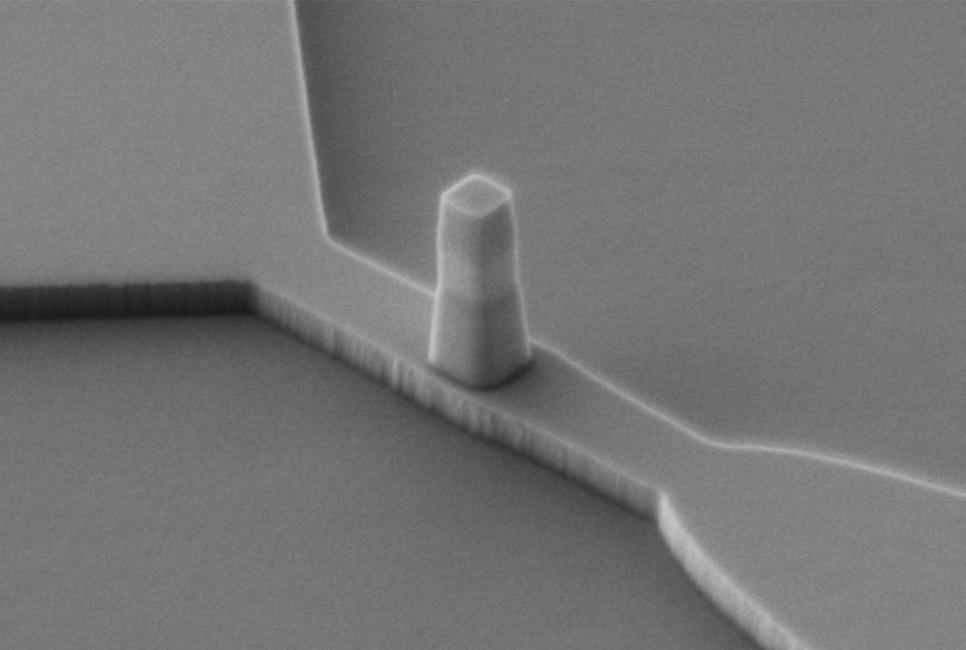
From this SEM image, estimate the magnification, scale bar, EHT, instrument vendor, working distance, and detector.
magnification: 23 K X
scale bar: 1000 nm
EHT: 2 kV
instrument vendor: JEOL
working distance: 17.1 mm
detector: LEI